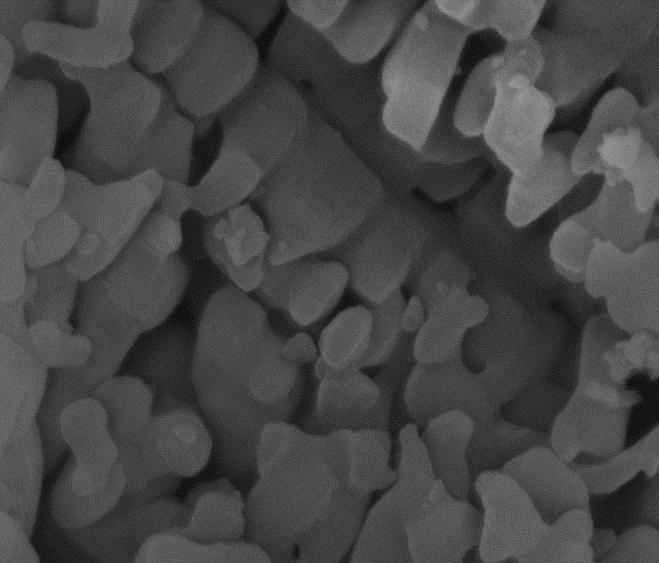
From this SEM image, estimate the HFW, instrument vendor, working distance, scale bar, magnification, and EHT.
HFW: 3.73 µm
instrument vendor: FEI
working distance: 5.5 mm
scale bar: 1000 nm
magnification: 80 K X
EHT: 10 kV